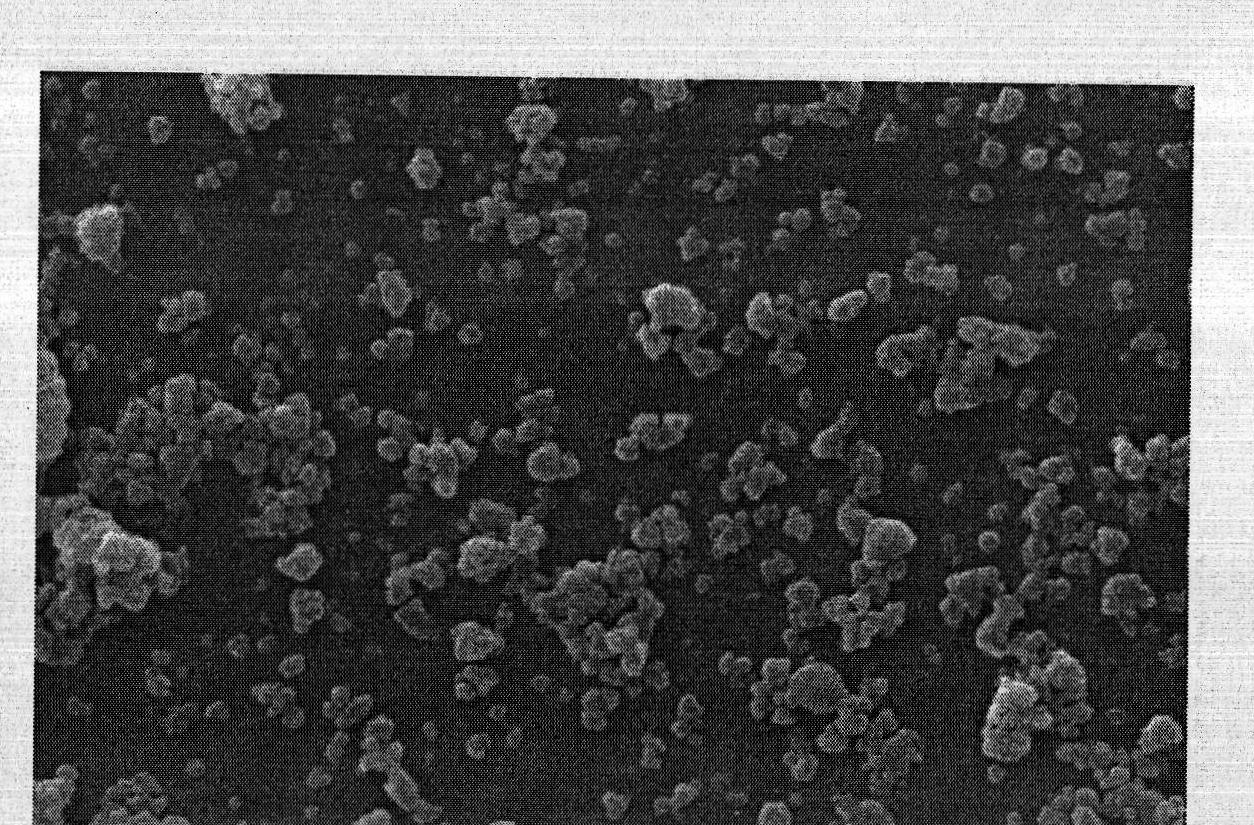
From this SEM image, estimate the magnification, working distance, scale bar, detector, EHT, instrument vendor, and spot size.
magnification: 10 K X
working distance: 14.8 mm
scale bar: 2000 nm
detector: SE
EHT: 25 kV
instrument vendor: FEI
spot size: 3